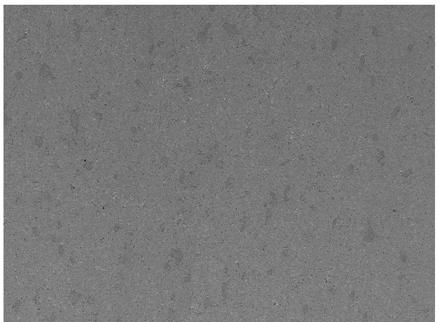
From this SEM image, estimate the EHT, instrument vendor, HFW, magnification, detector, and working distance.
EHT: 20 kV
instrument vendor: Tescan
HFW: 1040 µm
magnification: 0.2 K X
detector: SE, BSE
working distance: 16.85 mm